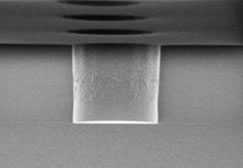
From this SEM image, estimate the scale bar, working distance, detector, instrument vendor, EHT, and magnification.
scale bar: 10000 nm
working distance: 10.4 mm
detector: SE(M)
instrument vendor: Hitachi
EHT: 0.8 kV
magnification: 4.5 K X